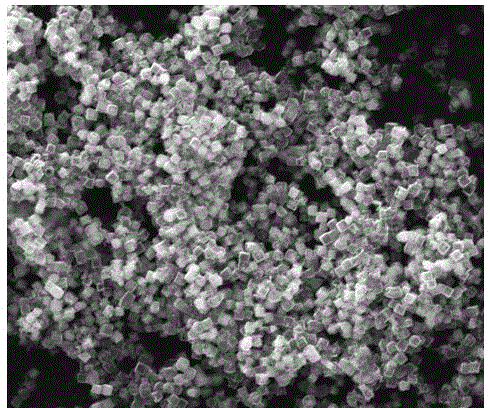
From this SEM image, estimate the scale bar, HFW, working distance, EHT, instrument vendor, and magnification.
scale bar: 10000 nm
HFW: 29.8 µm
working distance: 12.4 mm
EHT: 25 kV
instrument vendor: FEI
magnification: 10 K X